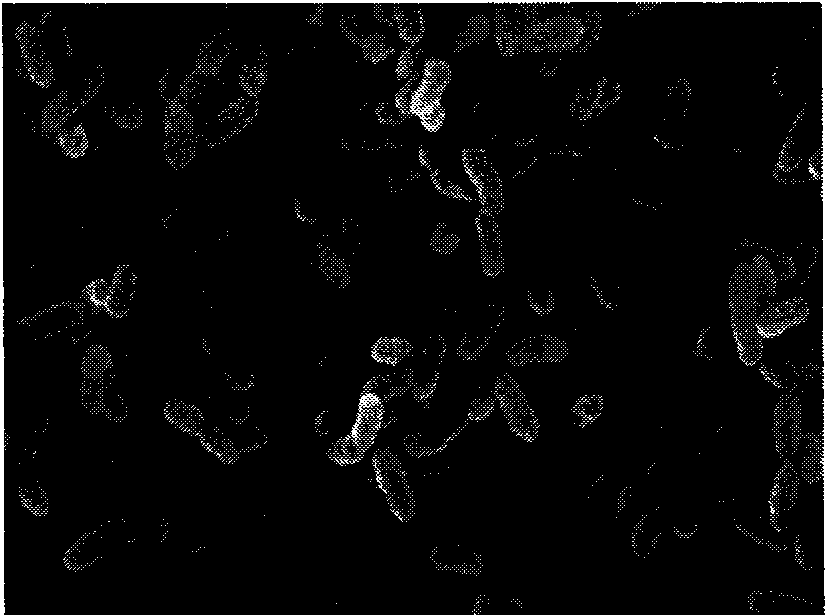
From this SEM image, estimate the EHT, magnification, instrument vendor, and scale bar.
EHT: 25 kV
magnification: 10 K X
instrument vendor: JEOL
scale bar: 1000 nm